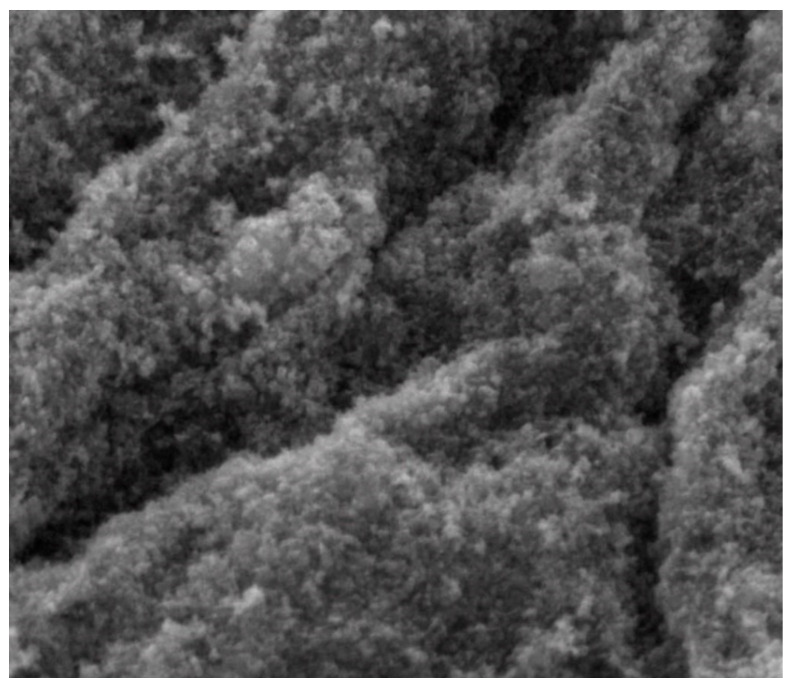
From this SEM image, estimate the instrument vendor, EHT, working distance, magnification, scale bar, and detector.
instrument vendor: FEI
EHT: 20 kV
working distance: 6.9 mm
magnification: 25 K X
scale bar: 4000 nm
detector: LFD